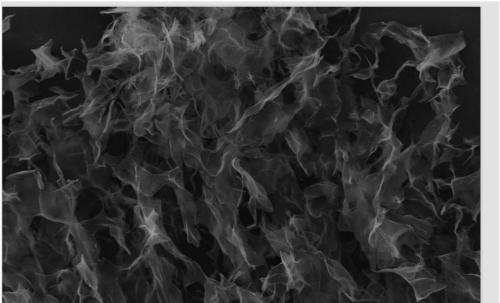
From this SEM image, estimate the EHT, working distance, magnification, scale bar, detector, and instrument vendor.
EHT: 10 kV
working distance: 7 mm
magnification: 1 K X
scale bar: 10000 nm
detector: SE1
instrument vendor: Zeiss